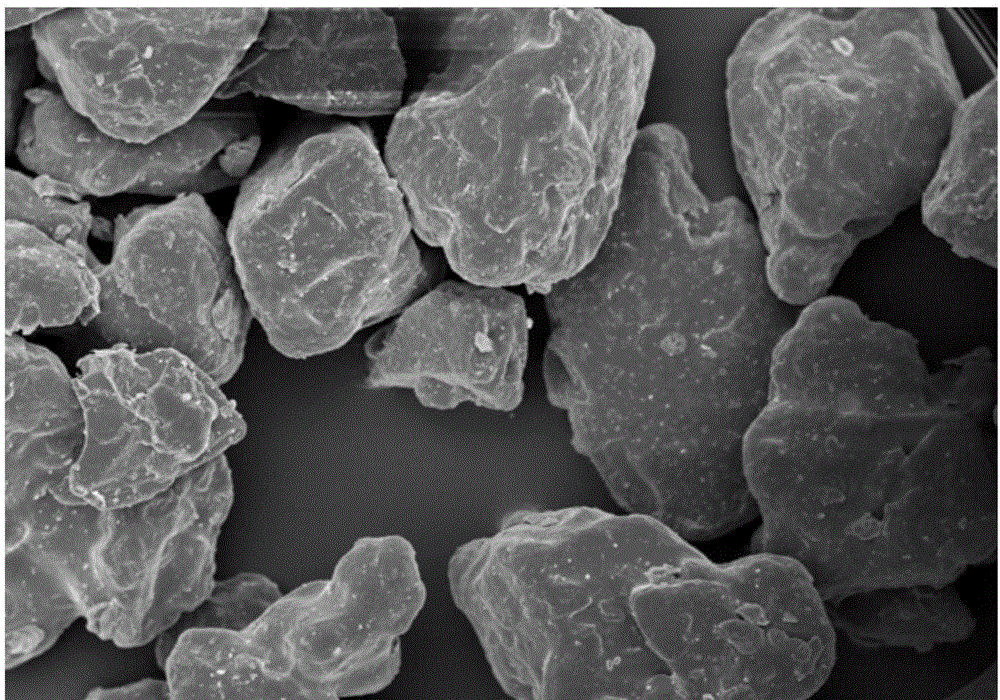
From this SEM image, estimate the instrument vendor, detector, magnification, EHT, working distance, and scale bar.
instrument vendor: Hitachi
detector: SE(M)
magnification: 0.4 K X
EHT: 10 kV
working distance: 7.5 mm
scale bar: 100000 nm